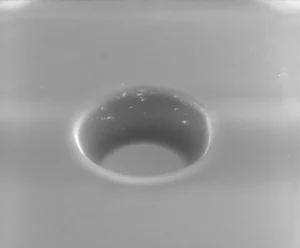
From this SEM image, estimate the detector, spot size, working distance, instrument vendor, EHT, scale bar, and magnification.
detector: LFD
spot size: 3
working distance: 10.7 mm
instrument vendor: FEI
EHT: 30 kV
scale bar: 50000 nm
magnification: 1.356 K X